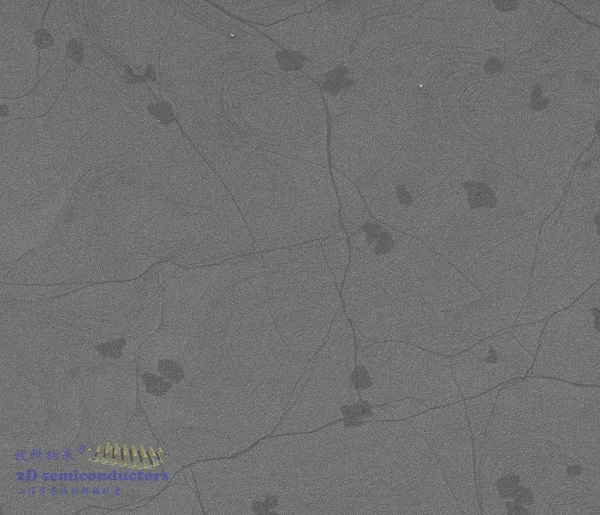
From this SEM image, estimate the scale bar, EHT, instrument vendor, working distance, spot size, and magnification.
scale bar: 10000 nm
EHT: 2 kV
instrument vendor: FEI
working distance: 12.5 mm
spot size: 2.5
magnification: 3 K X